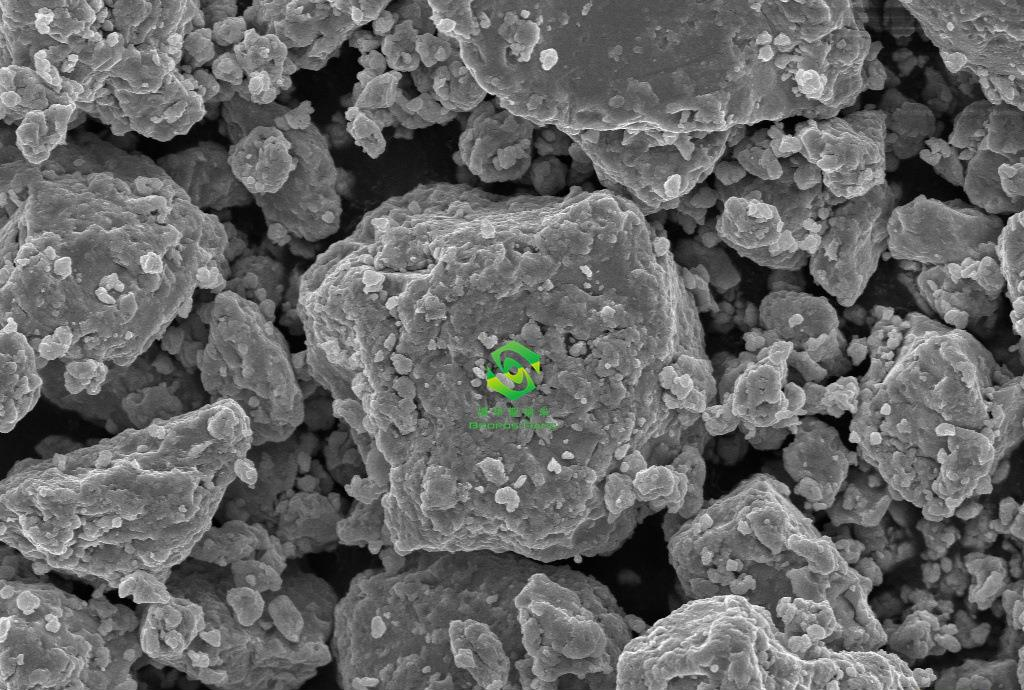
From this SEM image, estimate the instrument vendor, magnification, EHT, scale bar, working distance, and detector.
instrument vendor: Zeiss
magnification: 5 K X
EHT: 10 kV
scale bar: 2000 nm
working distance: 7.8 mm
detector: InLens